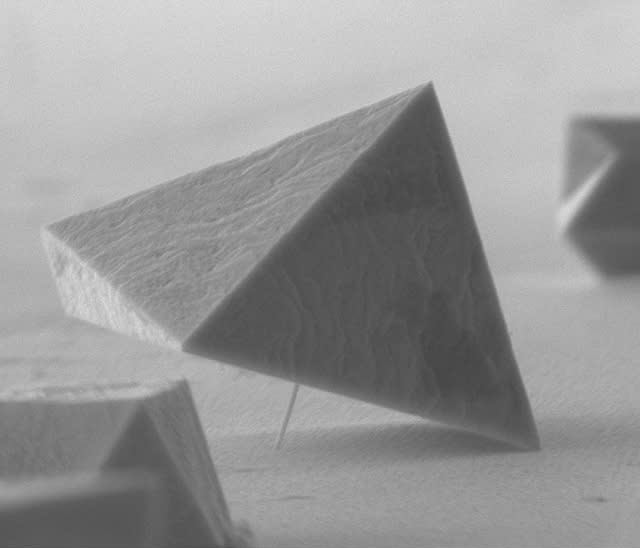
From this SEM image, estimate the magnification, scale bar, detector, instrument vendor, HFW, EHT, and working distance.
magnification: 50 K X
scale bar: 2000 nm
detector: ETD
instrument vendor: FEI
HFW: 5.97 µm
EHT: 10 kV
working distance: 9.5 mm